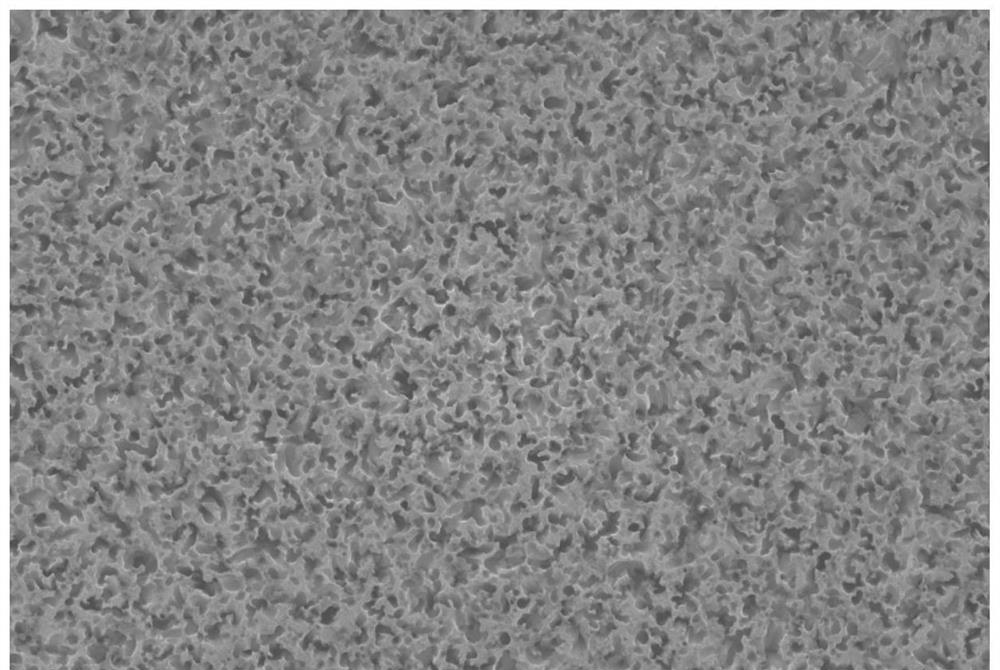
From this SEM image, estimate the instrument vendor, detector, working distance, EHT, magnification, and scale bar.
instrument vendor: Zeiss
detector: InLens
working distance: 3.8 mm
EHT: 10 kV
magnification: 10 K X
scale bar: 1000 nm